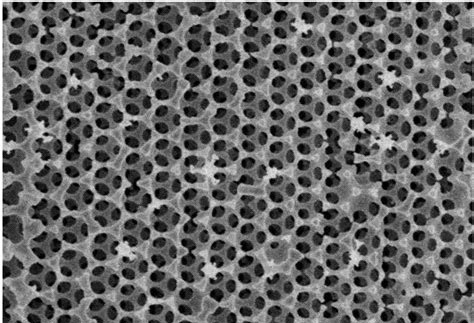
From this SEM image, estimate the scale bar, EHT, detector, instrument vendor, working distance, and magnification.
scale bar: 2000 nm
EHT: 3 kV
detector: SE(UL)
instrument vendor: Hitachi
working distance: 8.9 mm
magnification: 20 K X